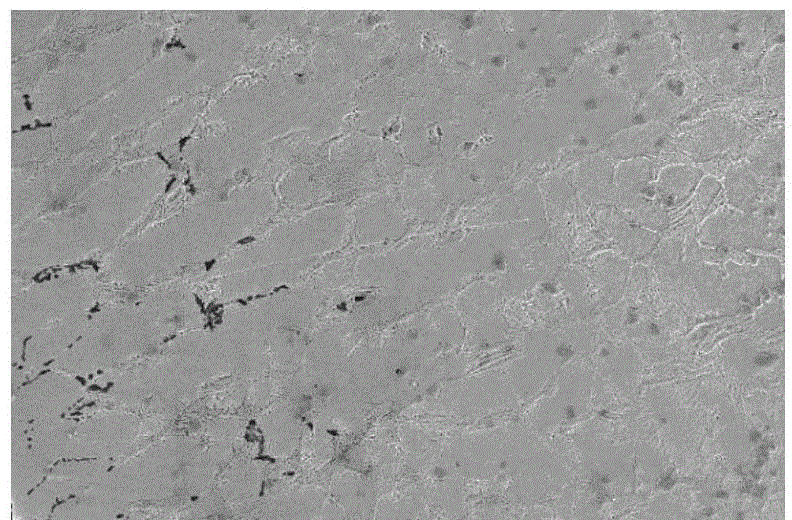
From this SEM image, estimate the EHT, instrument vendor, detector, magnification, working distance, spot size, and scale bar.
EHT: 10 kV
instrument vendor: FEI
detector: ETD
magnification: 2 K X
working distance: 4.7 mm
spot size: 4.5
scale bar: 40000 nm